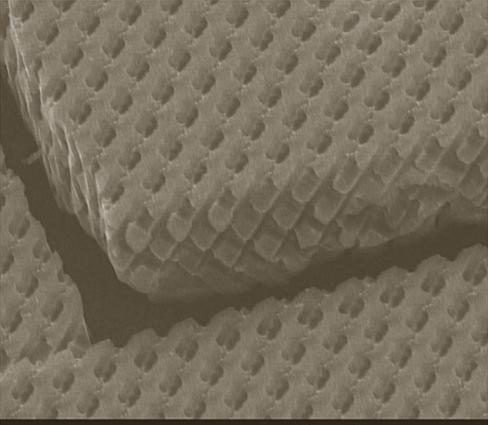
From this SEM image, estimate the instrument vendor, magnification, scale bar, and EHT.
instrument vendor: JEOL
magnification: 2.7 K X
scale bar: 5000 nm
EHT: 10 kV